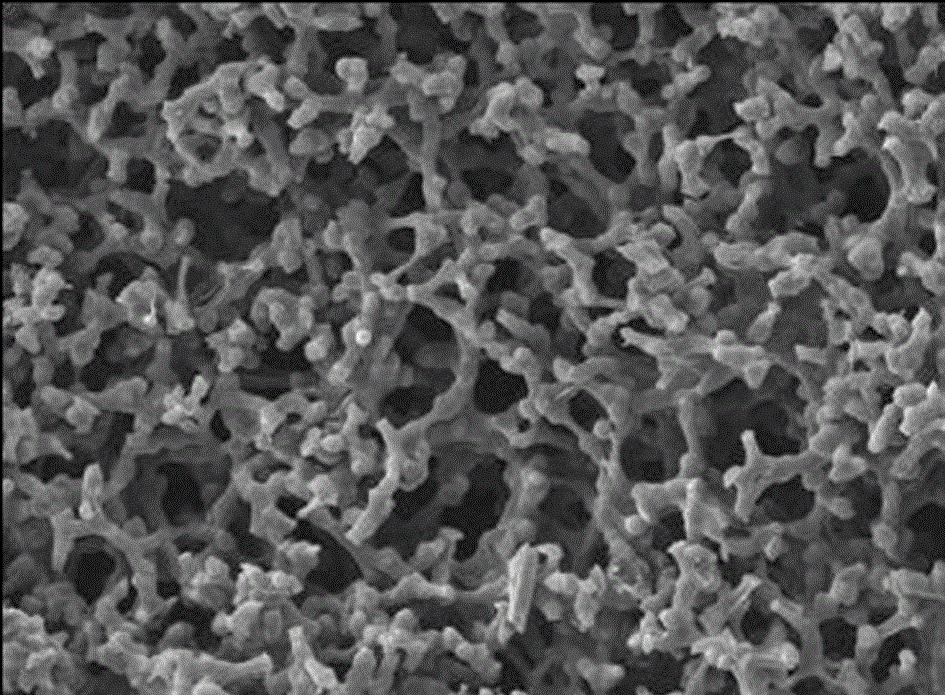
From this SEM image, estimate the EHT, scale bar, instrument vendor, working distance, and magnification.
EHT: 15 kV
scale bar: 10000 nm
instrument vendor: JEOL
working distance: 8 mm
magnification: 0.5 K X